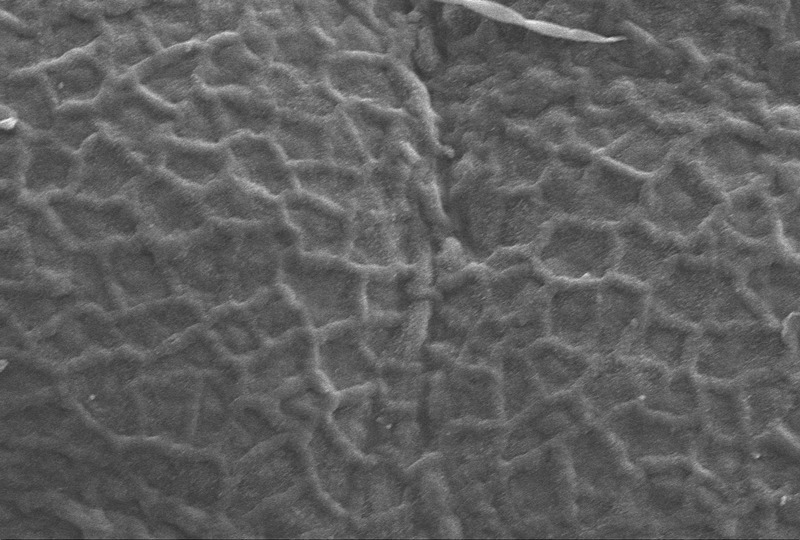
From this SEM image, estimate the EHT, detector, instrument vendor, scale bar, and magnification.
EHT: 17.13 kV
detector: SE1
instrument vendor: Zeiss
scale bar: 20000 nm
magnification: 1 K X